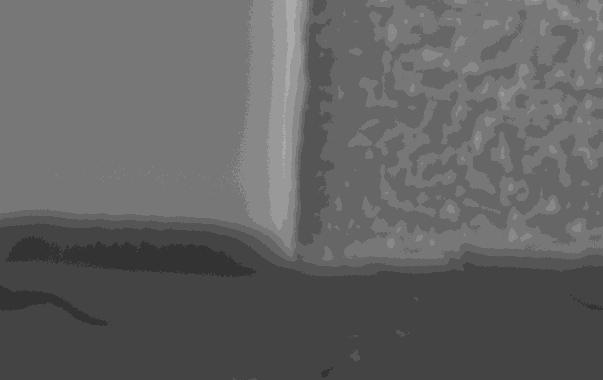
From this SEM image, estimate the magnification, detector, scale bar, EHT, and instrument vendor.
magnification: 15 K X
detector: SEI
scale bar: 2000 nm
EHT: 10 kV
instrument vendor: JEOL